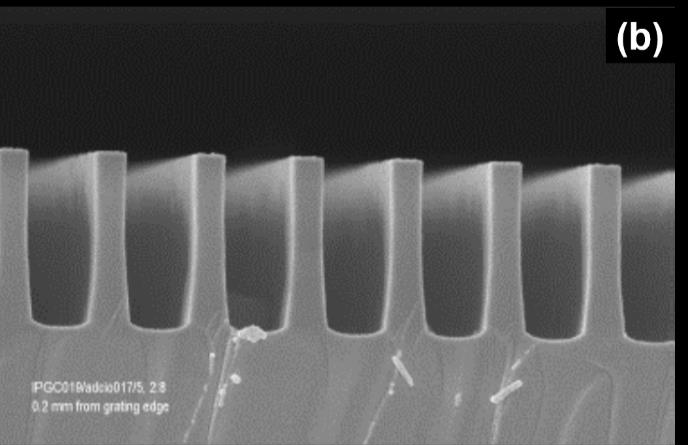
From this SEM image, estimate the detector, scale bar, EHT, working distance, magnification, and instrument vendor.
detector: InLens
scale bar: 1000 nm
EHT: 5 kV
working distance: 2 mm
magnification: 50 K X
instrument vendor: Zeiss